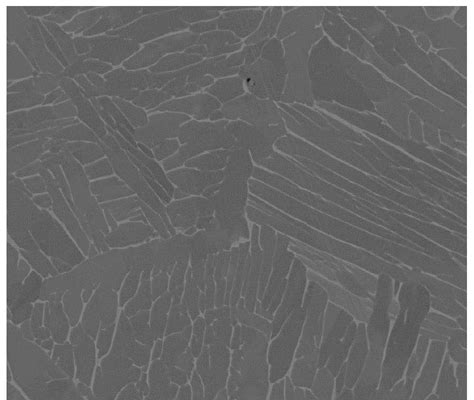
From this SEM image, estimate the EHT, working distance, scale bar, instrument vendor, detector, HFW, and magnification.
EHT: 20 kV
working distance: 4.7 mm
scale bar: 30000 nm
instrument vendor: FEI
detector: BSED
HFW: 120 µm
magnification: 2.5 K X